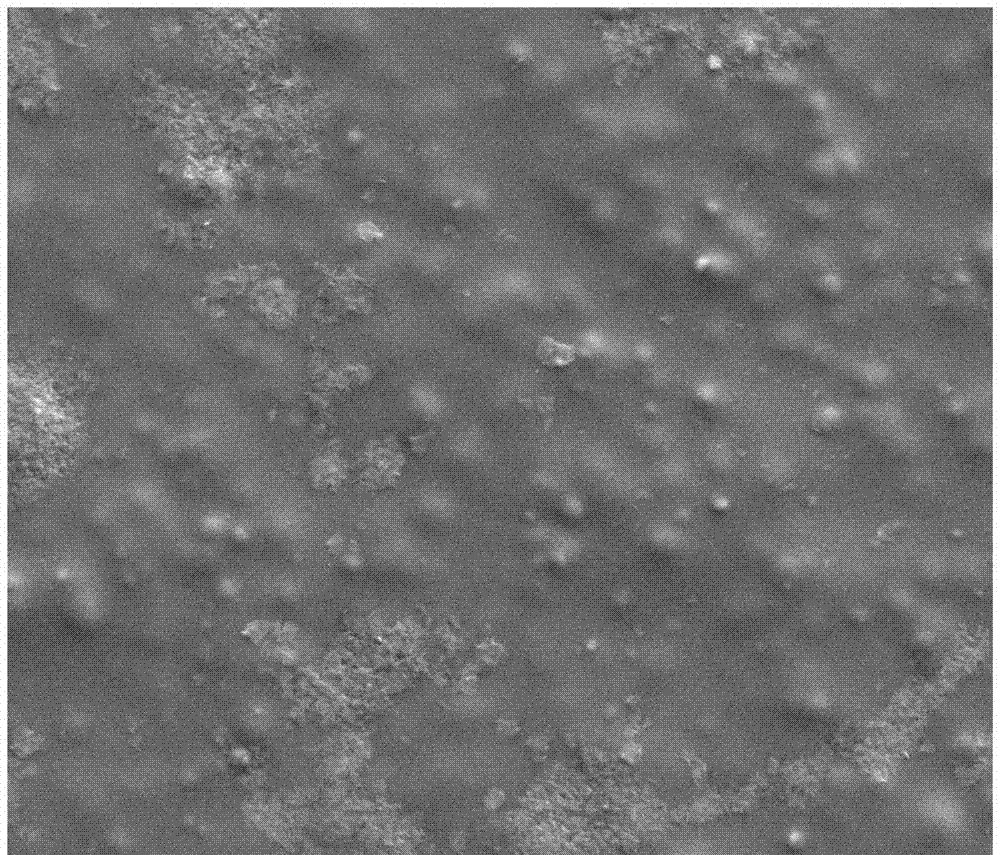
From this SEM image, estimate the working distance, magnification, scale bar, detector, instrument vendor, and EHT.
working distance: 8.8 mm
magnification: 2 K X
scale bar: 50000 nm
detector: ETD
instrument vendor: FEI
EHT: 10 kV